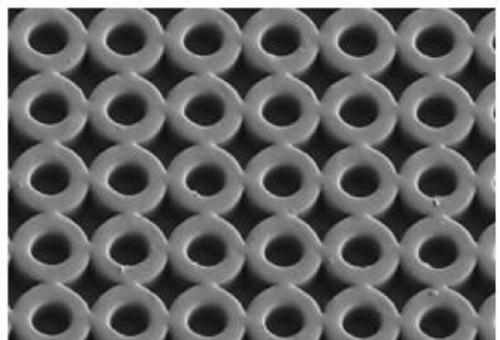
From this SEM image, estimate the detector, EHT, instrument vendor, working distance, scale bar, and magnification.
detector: SE(L)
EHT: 1 kV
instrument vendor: Hitachi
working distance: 8.8 mm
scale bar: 50000 nm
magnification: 1 K X